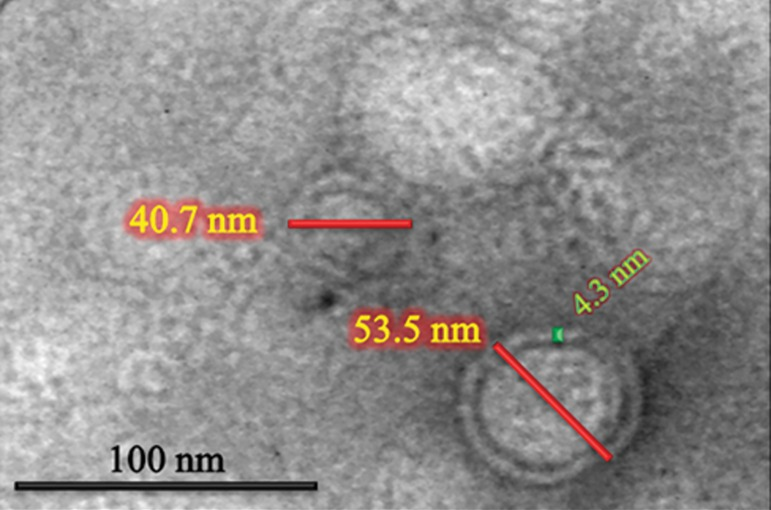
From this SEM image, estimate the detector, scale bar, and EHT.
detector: BF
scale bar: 30 nm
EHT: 150 kV